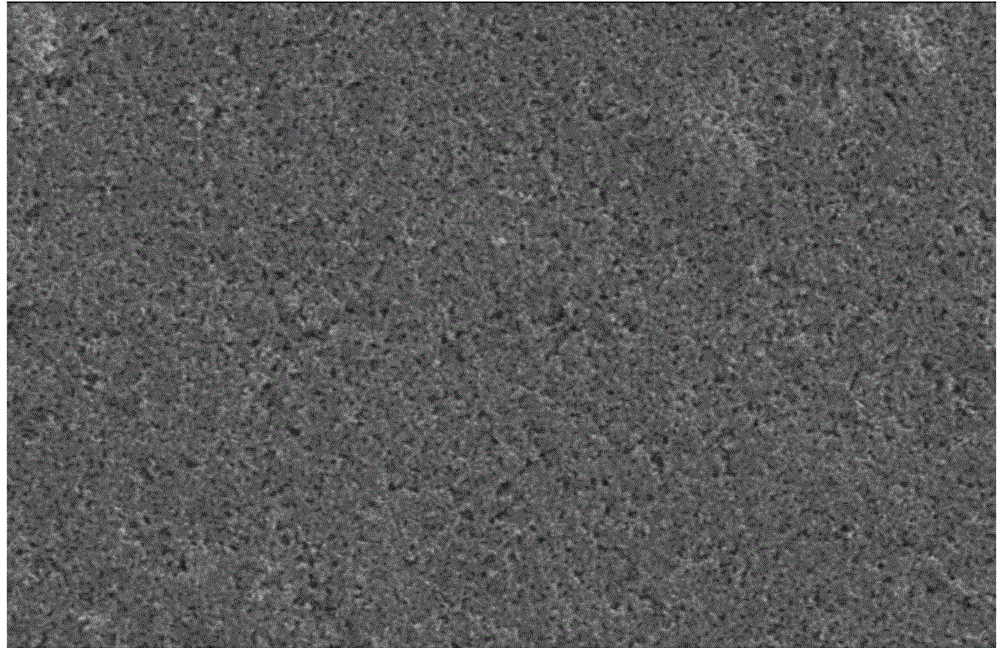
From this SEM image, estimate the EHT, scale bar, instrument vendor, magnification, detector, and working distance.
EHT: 20 kV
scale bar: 50000 nm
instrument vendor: Hitachi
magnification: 1 K X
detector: SE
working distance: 4.6 mm